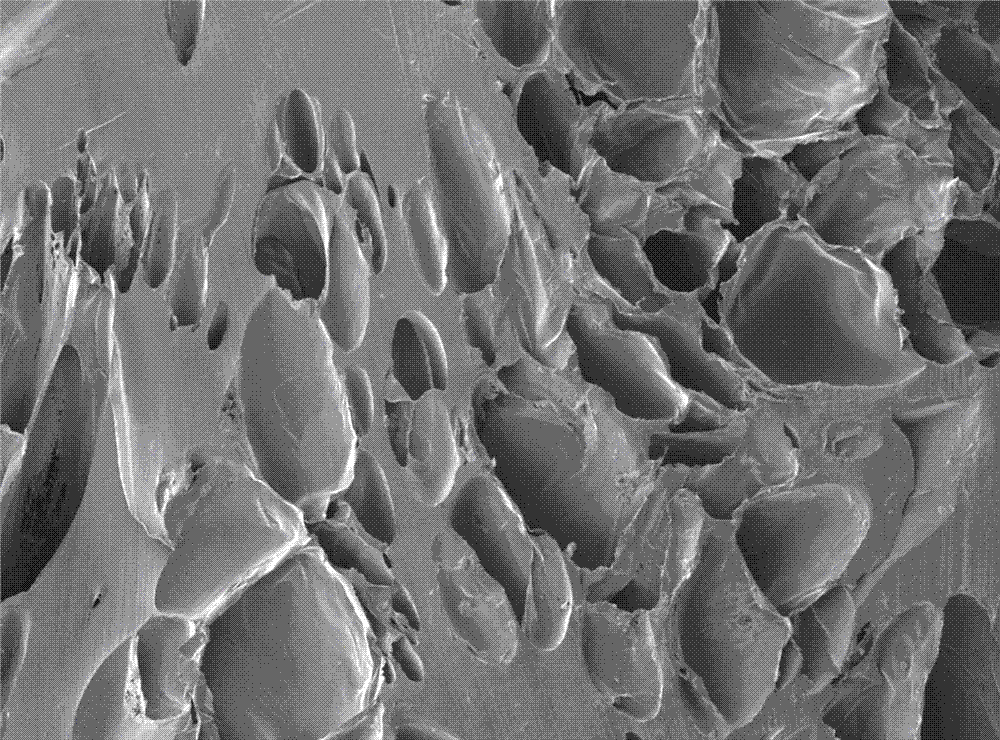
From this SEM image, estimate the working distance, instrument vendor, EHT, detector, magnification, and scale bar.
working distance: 8 mm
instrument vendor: JEOL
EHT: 3 kV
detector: SEI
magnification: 0.05 K X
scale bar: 100000 nm